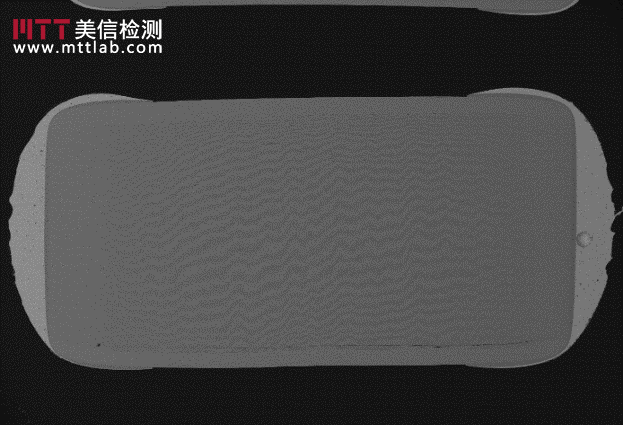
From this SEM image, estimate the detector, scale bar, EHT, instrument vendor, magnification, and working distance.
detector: BSE3D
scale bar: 1000000 nm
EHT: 15 kV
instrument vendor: Hitachi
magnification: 0.035 K X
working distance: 10.4 mm